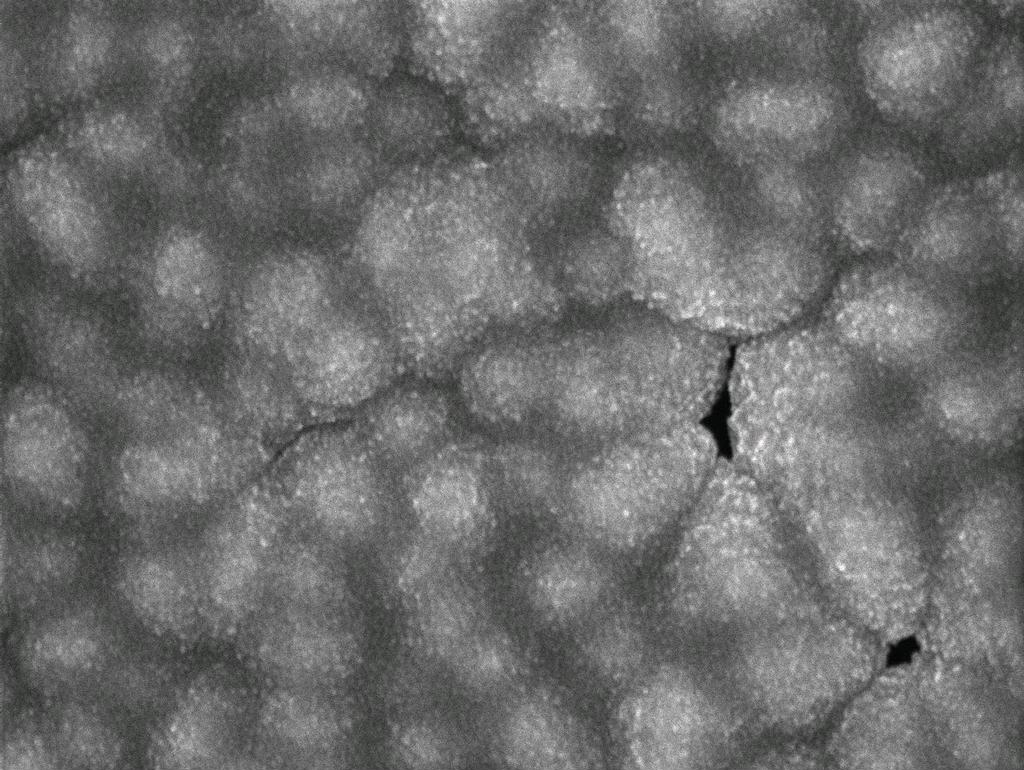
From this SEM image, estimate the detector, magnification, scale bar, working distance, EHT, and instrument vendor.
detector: SEI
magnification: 40 K X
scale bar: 100 nm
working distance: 8.5 mm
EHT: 5 kV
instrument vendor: JEOL